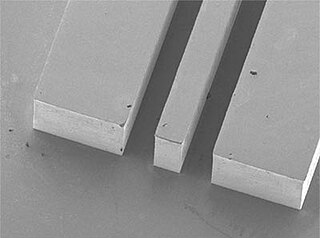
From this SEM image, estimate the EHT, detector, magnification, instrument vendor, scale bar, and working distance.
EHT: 5 kV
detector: LOWER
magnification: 0.037 K X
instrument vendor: JEOL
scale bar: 100000 nm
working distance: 14.9 mm